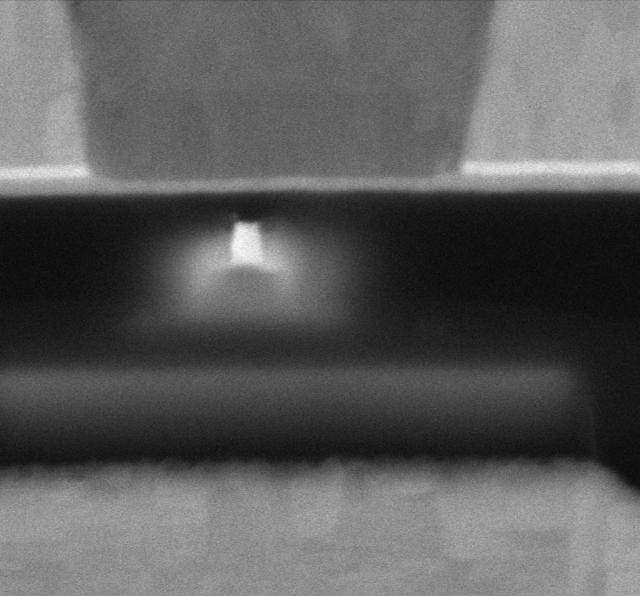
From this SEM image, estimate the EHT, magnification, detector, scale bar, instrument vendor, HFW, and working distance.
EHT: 5 kV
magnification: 568.609 K X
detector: MD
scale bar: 100 nm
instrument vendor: Thermo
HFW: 0.635 µm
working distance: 2 mm